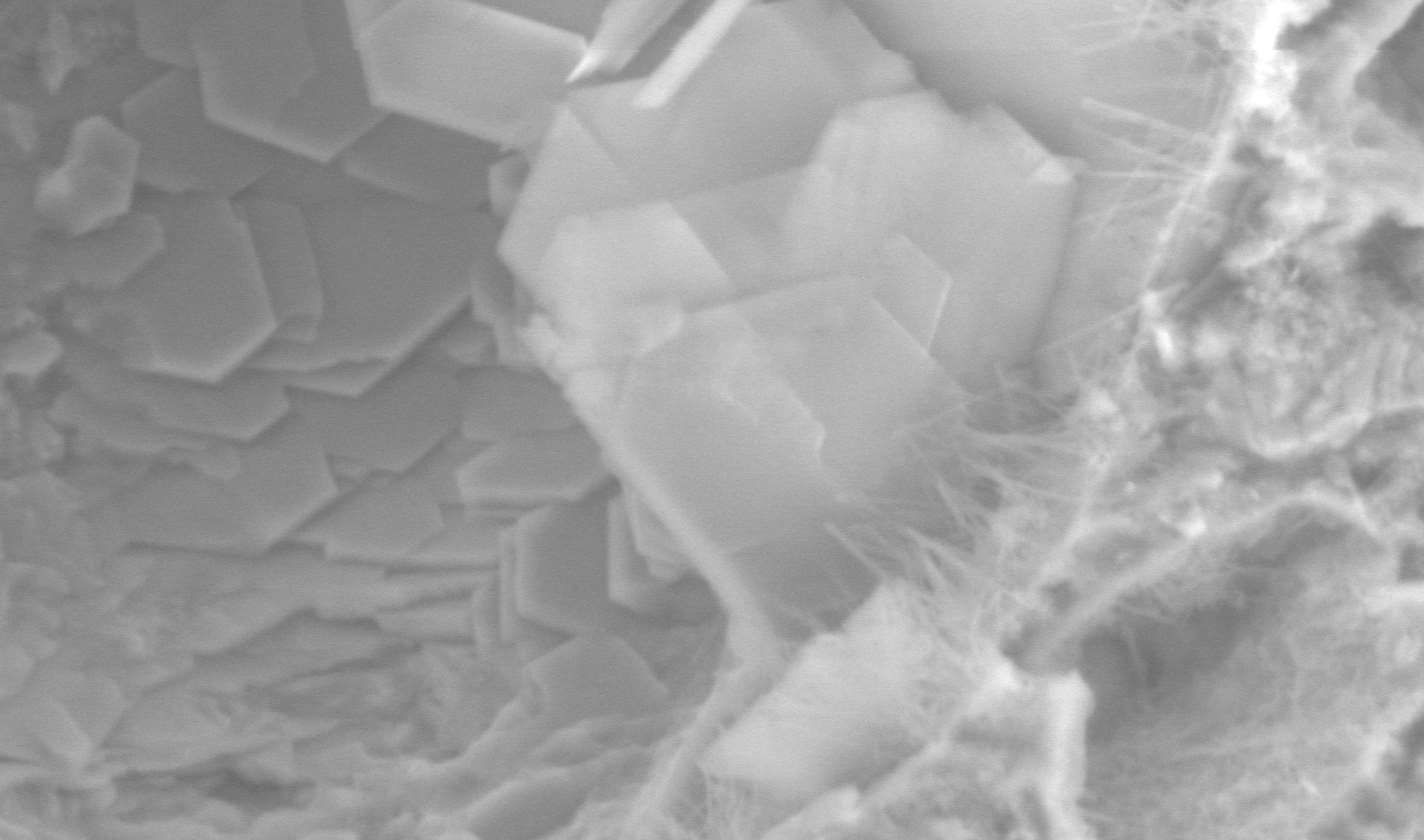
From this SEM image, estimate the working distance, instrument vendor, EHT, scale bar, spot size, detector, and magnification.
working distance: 11.5 mm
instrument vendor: FEI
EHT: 10 kV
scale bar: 10000 nm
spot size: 5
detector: GSE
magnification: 3 K X